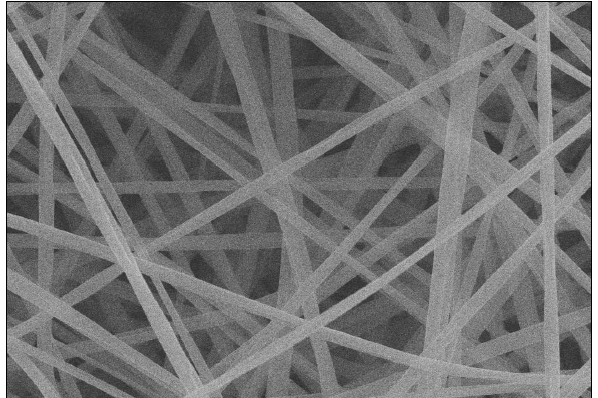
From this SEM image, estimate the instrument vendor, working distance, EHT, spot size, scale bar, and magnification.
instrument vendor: other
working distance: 5 mm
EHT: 20 kV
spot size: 8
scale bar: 15000 nm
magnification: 2 K X